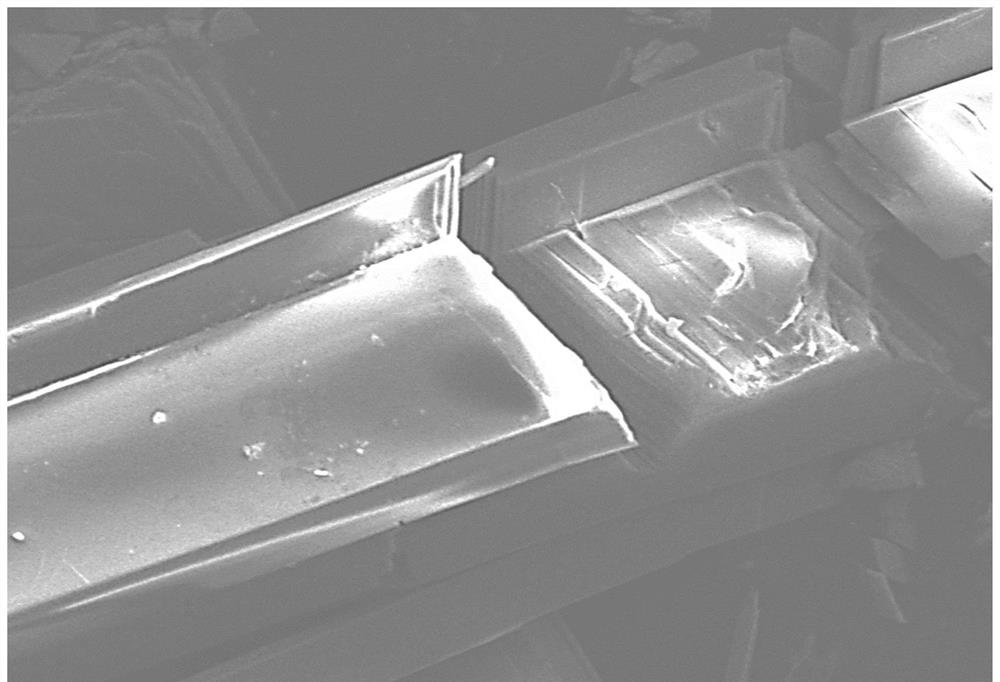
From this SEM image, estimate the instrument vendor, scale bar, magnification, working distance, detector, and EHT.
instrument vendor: JEOL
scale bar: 10000 nm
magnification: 1 K X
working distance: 14.9 mm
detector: LED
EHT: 10 kV